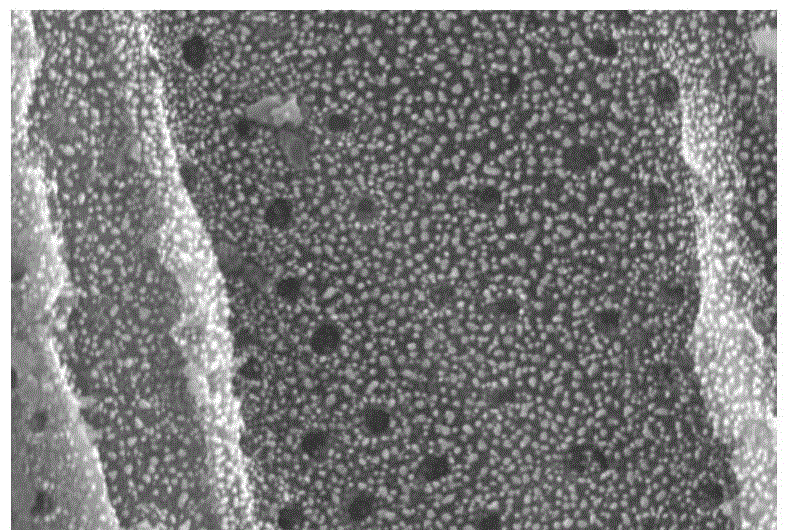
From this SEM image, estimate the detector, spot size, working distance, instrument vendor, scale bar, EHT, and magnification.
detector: SEI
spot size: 30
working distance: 11 mm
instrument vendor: JEOL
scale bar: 5000 nm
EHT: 20 kV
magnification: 5 K X